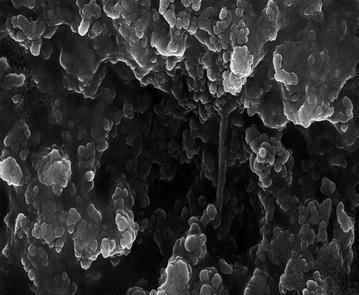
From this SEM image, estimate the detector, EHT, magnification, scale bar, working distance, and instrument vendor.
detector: InLens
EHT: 20 kV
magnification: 75 K X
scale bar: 200 nm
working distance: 4.2 mm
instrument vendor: Zeiss